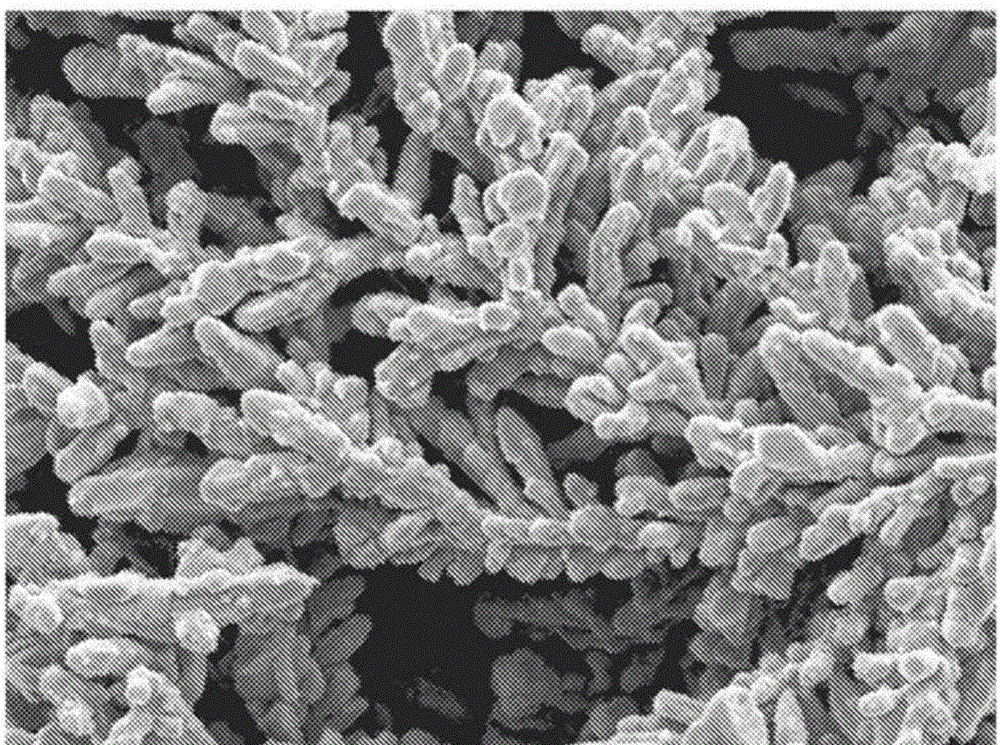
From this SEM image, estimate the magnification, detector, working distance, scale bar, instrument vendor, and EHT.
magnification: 10 K X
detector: SEI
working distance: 11.9 mm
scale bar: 1000 nm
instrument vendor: JEOL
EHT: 5 kV